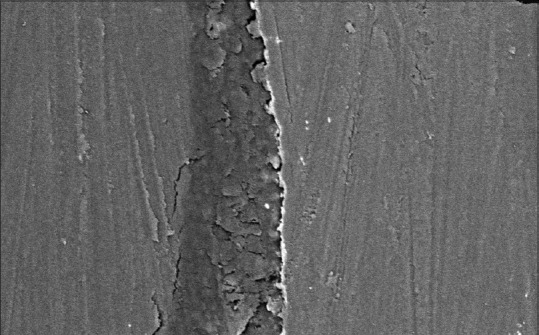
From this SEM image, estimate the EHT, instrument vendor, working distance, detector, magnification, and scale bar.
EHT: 20 kV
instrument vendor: Zeiss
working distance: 13.5 mm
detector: NTS BSD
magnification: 1 K X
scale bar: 20000 nm